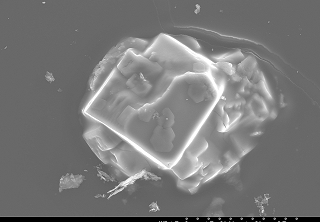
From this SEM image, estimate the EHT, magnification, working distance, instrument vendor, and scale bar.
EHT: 15 kV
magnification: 1.8 K X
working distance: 15 mm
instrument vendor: JEOL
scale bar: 25000 nm